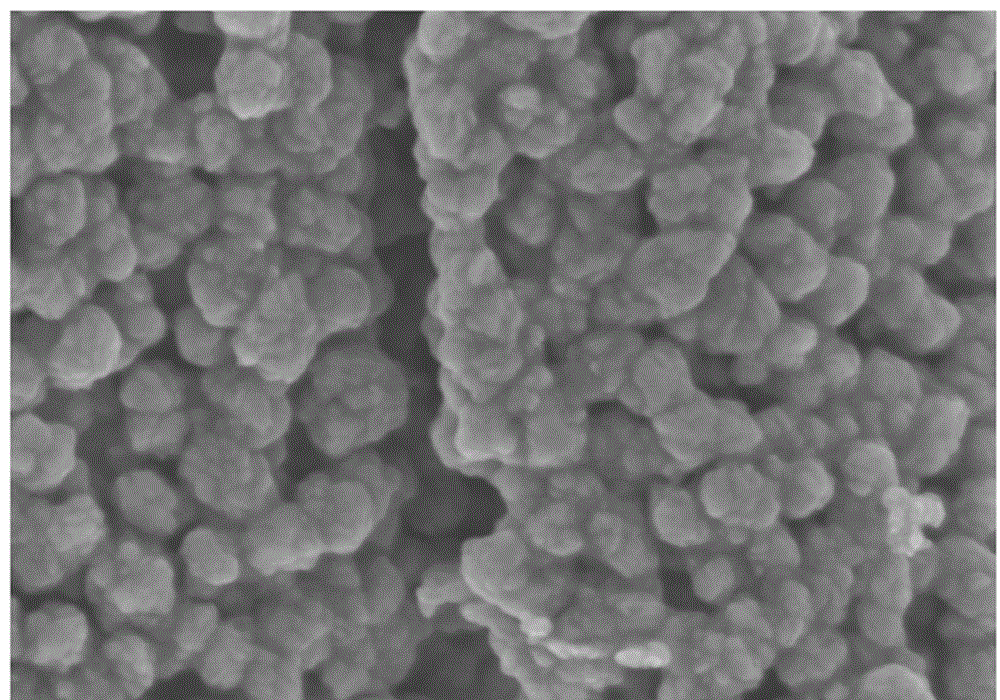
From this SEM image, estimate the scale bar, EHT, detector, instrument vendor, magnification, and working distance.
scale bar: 500 nm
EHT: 3 kV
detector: SE(M)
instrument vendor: Hitachi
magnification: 100 K X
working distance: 4.2 mm